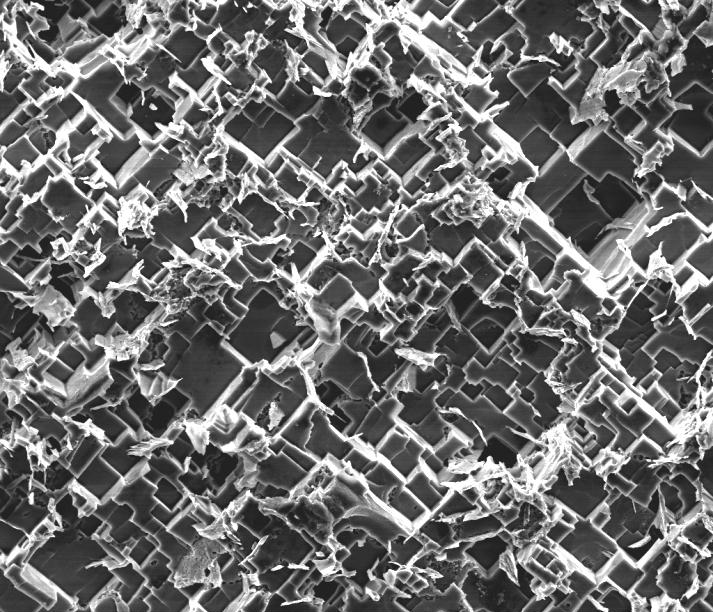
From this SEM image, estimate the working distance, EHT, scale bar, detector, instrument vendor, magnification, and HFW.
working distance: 17.6 mm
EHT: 10 kV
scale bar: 40000 nm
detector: ETD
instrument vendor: FEI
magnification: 2 K X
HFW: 128 µm